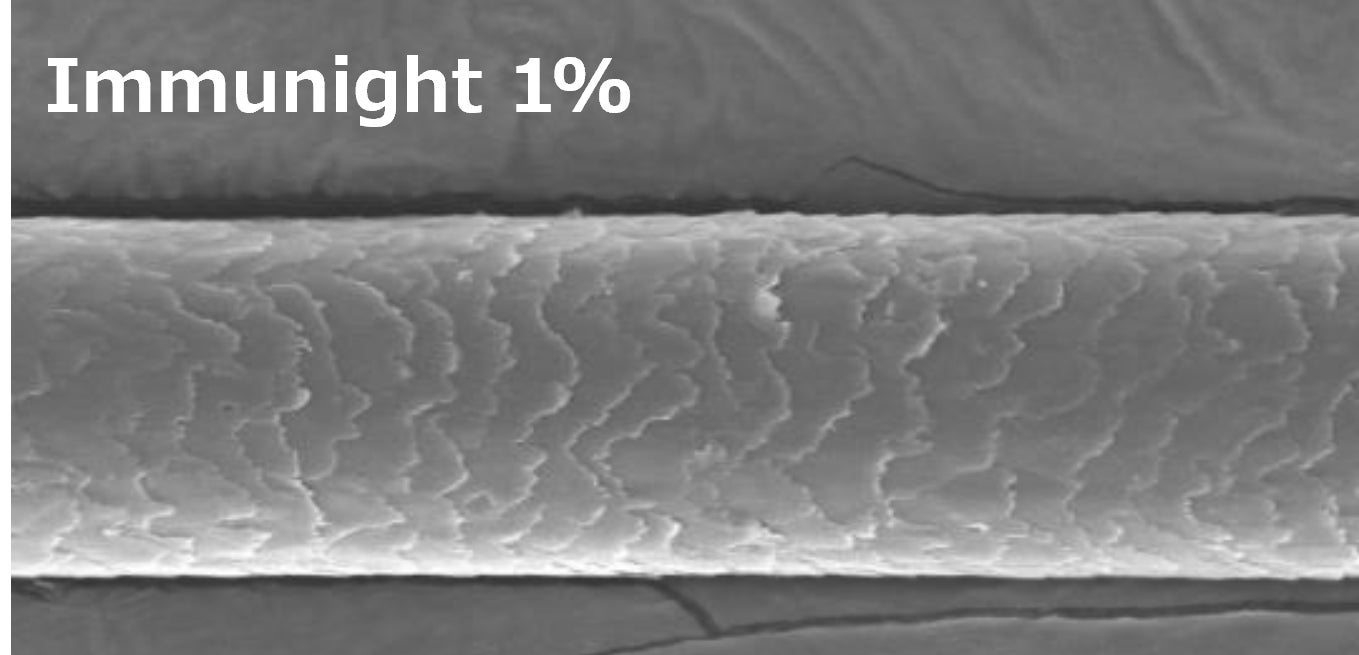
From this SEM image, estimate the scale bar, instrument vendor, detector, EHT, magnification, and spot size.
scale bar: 50000 nm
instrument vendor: JEOL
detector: SE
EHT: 15 kV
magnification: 1 K X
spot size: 8